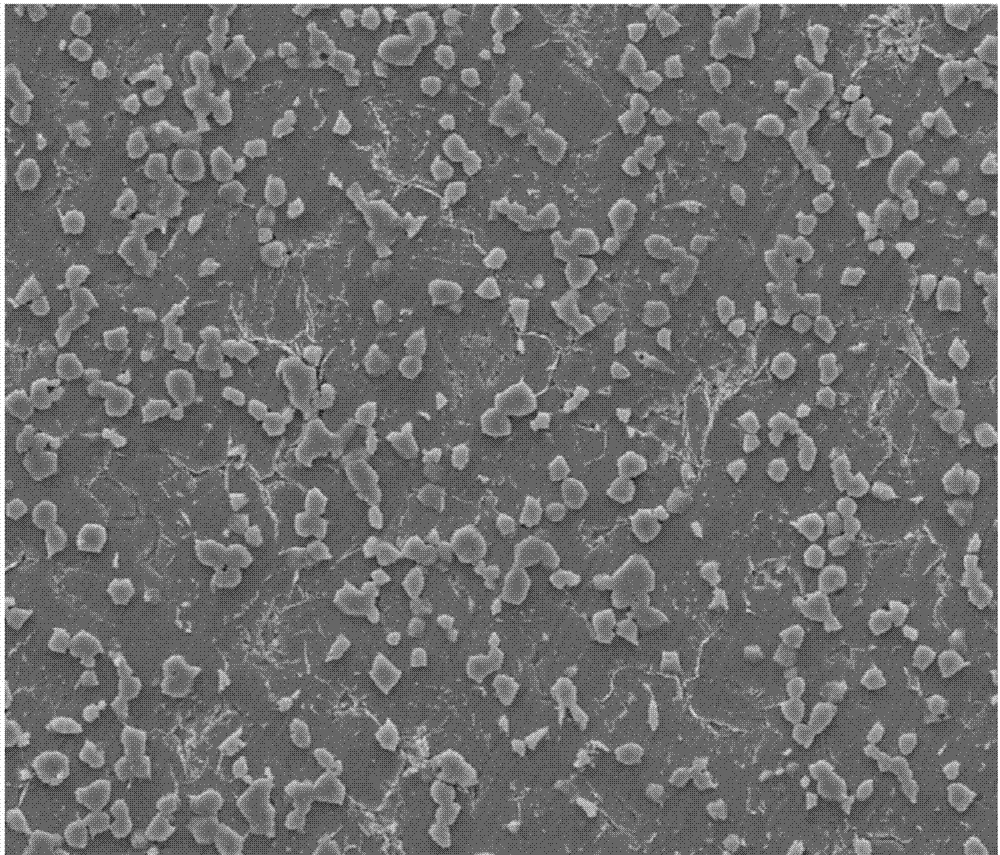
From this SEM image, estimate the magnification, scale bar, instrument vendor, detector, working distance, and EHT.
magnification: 2 K X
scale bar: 50000 nm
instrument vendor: FEI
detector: ETD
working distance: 8.3 mm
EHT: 20 kV